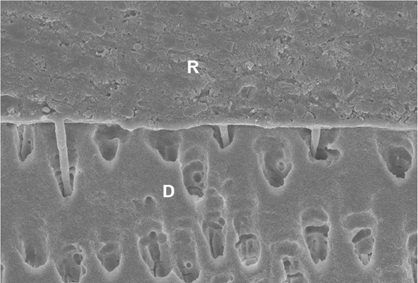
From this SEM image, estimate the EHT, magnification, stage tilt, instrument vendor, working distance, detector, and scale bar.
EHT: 10 kV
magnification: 5 K X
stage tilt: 57.5°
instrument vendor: Zeiss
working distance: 5.1 mm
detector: InLens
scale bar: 2000 nm